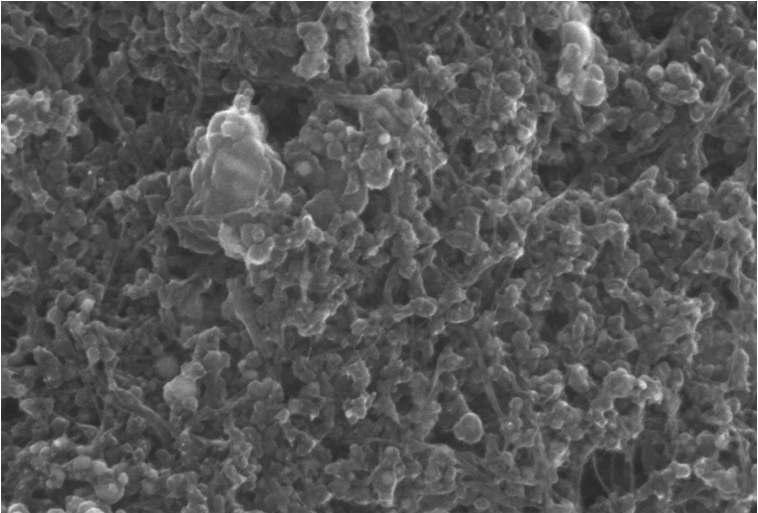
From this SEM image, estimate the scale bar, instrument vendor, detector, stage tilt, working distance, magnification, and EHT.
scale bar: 100 nm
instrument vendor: Zeiss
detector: InLens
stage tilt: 59.9°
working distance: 4.8 mm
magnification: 200 K X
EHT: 10 kV